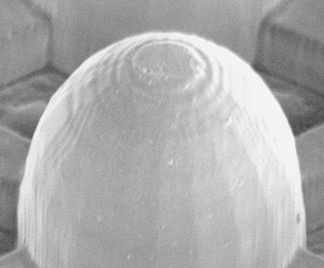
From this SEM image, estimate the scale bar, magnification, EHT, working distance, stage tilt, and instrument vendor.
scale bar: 10000 nm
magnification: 3.363 K X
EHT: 5 kV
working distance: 10.3 mm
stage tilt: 0°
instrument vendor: FEI